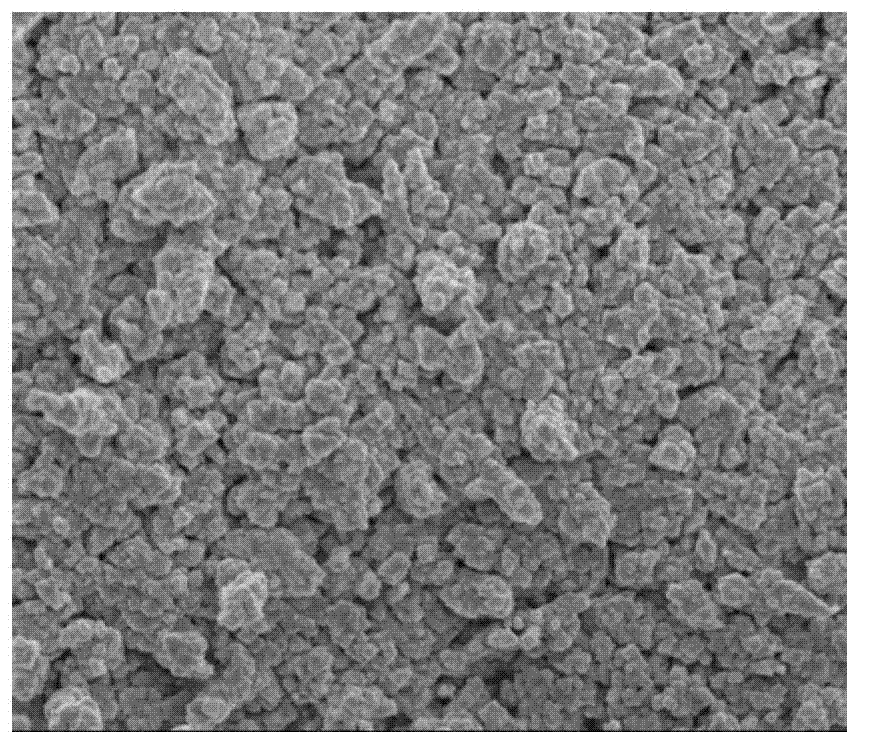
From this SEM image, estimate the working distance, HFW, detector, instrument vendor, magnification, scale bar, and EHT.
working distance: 5.9 mm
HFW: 8 µm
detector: TLD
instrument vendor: FEI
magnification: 30 K X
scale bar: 2000 nm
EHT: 5 kV